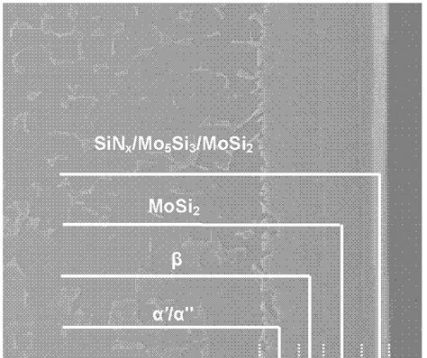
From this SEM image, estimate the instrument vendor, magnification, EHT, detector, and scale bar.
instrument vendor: FEI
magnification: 1.2 K X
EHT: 20 kV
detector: SE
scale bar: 50000 nm